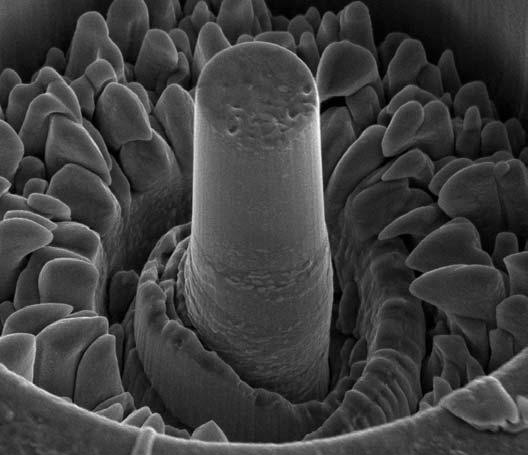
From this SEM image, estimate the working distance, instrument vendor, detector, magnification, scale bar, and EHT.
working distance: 9.8 mm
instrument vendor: FEI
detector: ETD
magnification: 12.5 K X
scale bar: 5000 nm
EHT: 5 kV